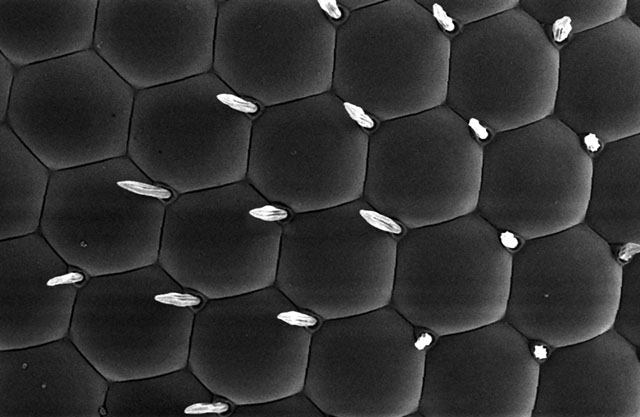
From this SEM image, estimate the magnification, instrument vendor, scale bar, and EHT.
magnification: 1.5 K X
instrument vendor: JEOL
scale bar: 10000 nm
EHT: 5 kV